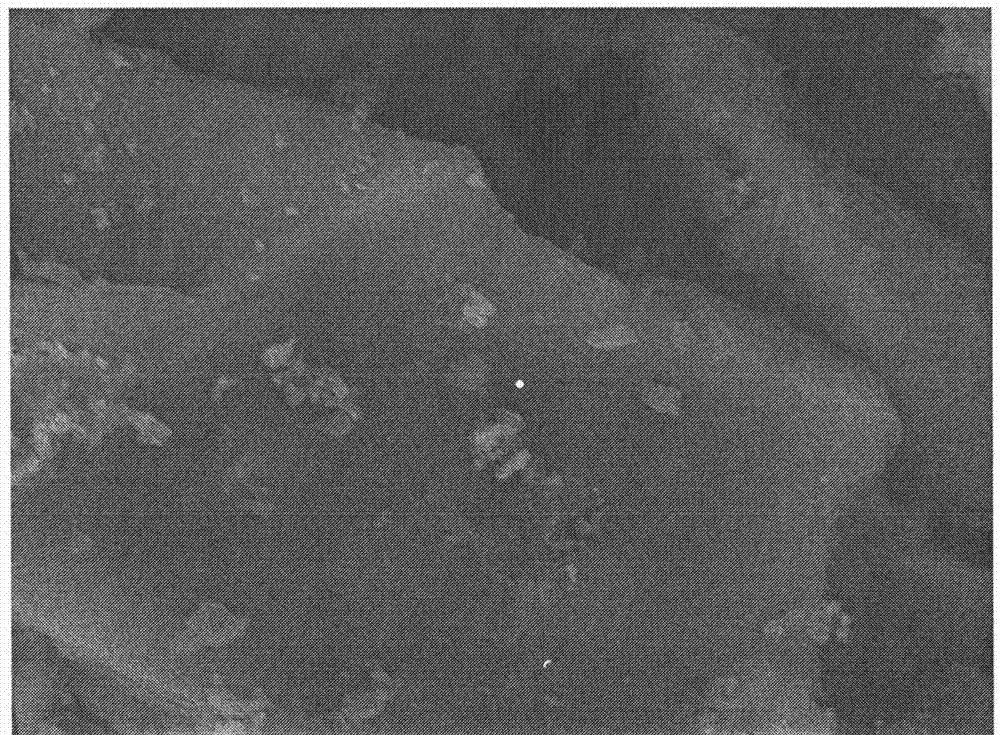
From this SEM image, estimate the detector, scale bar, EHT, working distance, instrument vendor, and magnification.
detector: LEI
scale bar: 1000 nm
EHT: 15 kV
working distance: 12.4 mm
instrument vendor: JEOL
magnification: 6 K X